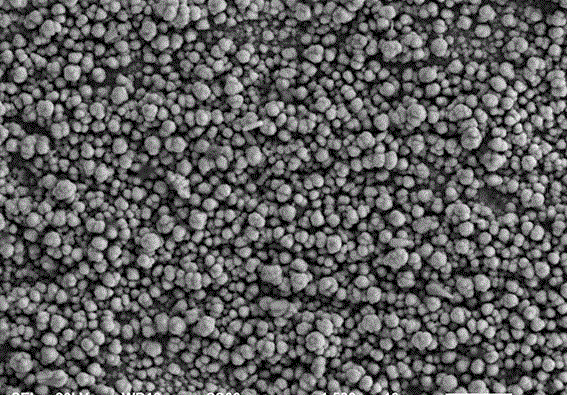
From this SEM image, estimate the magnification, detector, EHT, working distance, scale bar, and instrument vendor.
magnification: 1.5 K X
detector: SEI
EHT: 20 kV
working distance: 13 mm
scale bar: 10000 nm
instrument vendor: JEOL